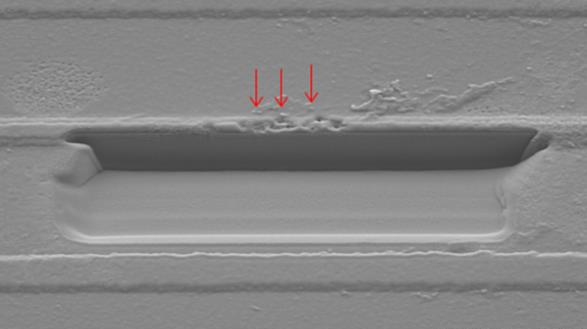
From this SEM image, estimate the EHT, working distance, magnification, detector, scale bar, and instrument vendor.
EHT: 5 kV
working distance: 18.1 mm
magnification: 3 K X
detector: SE(M)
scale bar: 10000 nm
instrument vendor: Hitachi